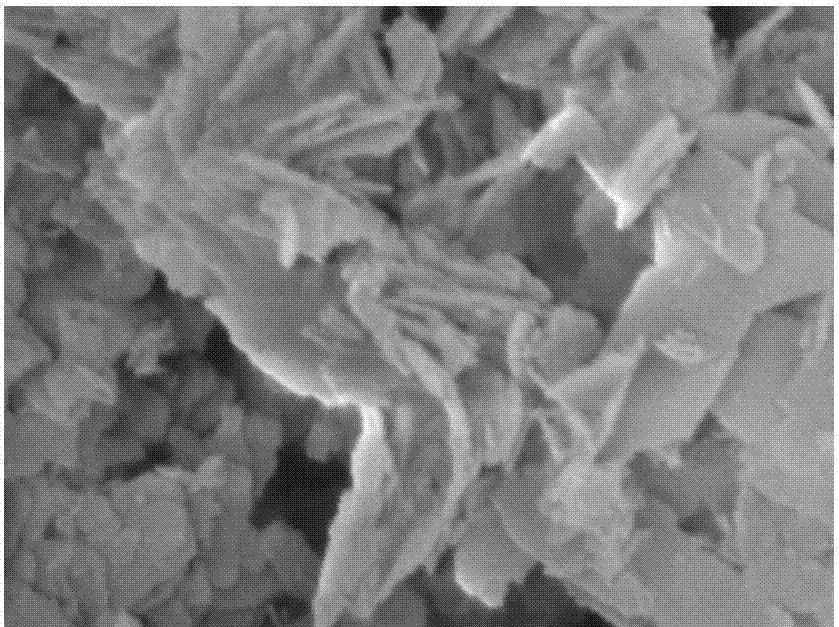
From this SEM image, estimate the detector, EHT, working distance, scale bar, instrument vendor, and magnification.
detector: SEI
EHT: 3 kV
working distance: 22.5 mm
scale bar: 100 nm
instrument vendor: JEOL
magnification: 50 K X